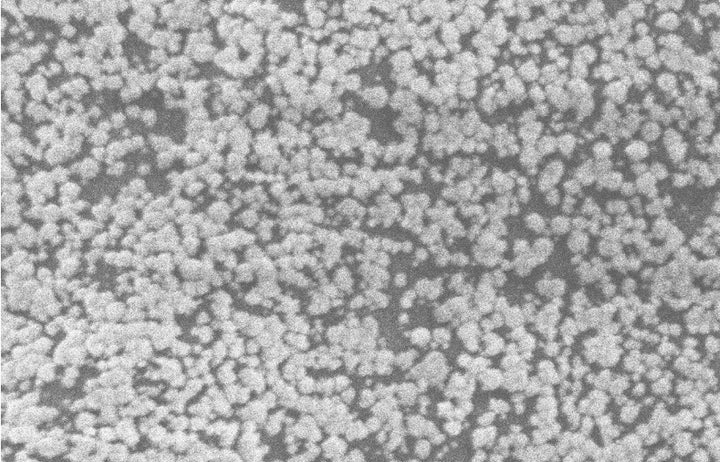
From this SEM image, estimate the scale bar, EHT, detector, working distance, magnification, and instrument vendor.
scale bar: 2000 nm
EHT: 5 kV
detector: SE2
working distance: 8.6 mm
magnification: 32.49 K X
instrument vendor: Zeiss